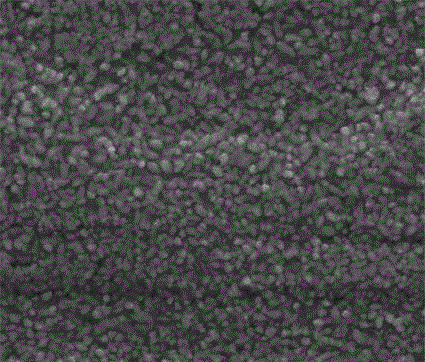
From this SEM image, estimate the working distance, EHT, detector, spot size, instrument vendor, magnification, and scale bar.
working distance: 10 mm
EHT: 20 kV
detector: ETD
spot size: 4.5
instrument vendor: FEI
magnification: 5 K X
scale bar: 10000 nm